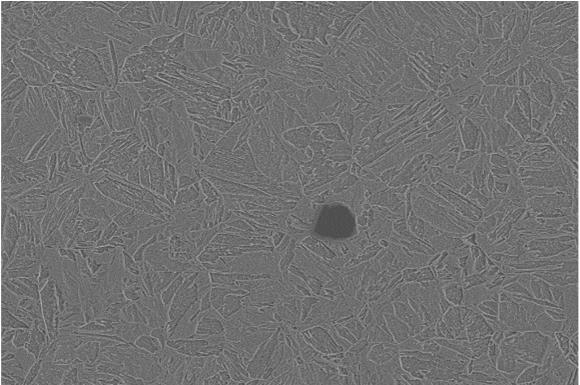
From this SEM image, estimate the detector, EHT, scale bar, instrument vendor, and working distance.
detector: SE2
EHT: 4 kV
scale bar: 1000 nm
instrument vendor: Zeiss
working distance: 8.5 mm